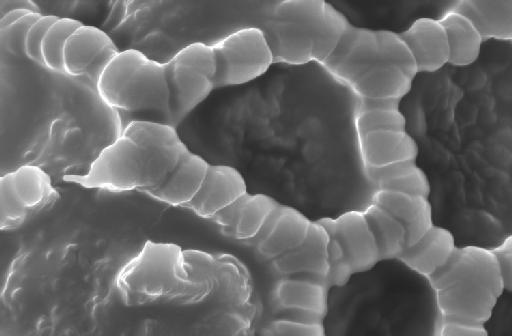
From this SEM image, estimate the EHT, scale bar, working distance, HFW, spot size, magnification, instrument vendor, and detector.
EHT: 10 kV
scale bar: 5000 nm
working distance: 20.7 mm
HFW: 18.1 µm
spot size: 2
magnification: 3.871 K X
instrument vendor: FEI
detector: ETD